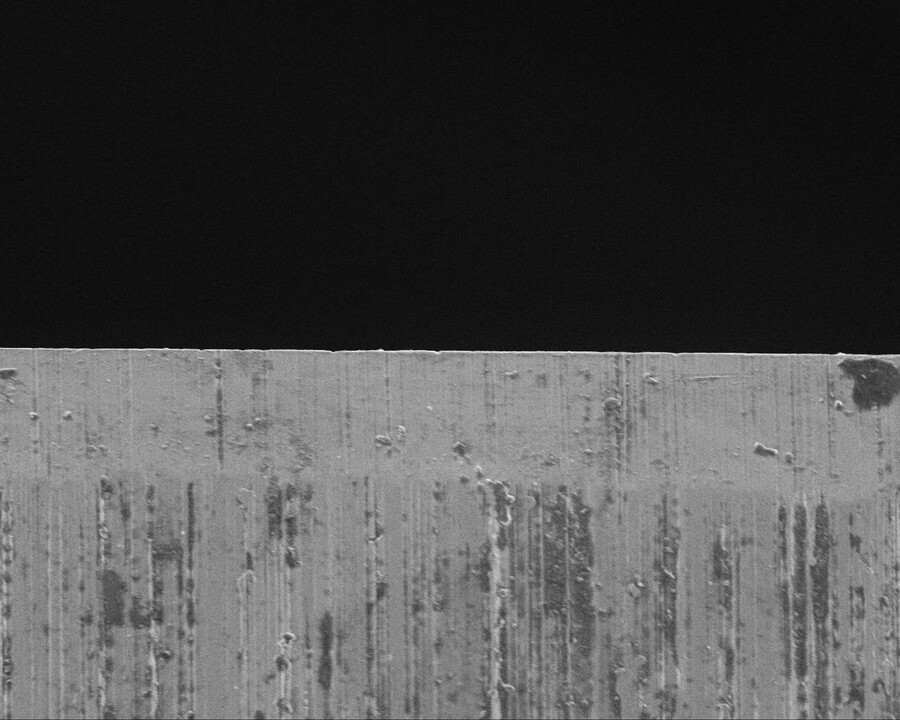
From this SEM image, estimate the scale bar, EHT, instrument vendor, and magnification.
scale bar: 100000 nm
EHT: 10 kV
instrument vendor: JEOL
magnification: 0.24 K X